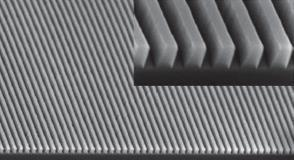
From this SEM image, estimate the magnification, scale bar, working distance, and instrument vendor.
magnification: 35.46 K X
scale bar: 1000 nm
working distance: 5 mm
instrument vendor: Zeiss